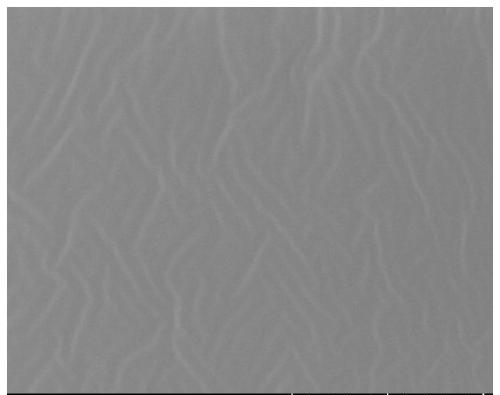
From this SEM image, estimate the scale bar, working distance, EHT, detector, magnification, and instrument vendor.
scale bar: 100000 nm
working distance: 8.5 mm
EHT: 3 kV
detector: SE(UL)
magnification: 0.5 K X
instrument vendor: Hitachi